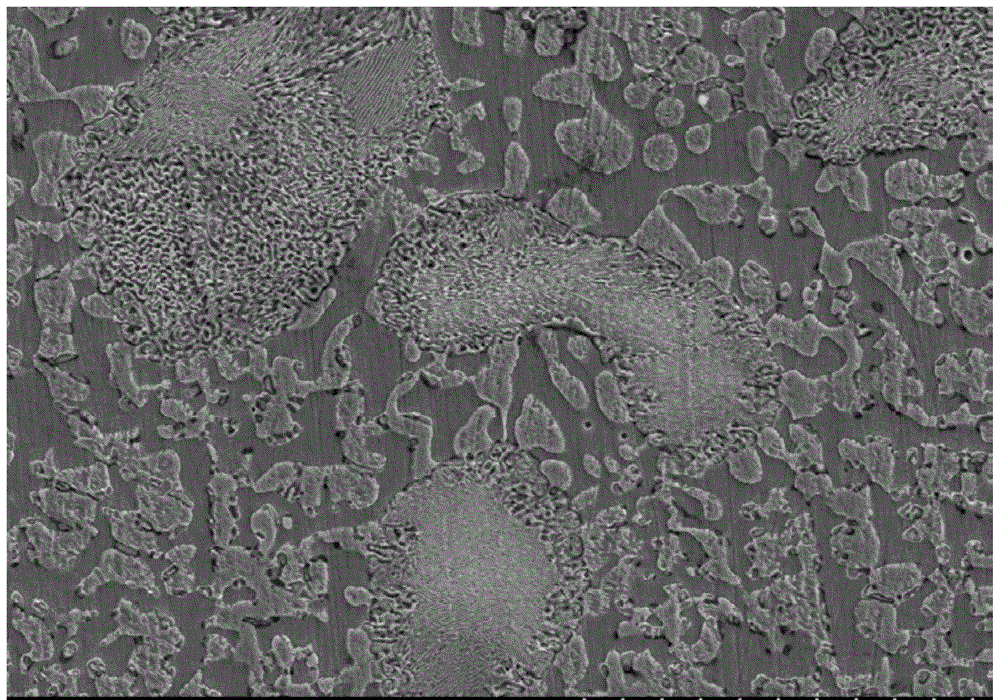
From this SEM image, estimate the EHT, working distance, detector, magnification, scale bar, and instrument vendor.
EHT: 20 kV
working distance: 11.3 mm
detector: SE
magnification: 1 K X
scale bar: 50000 nm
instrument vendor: Hitachi